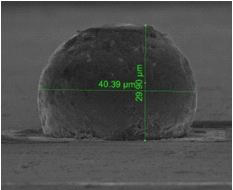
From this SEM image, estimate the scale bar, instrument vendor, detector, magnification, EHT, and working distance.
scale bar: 30000 nm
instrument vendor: FEI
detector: SE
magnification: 5 K X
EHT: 10 kV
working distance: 15.5 mm